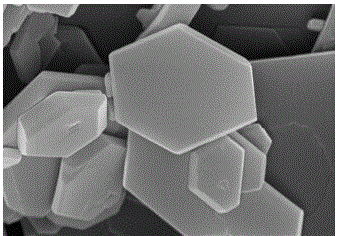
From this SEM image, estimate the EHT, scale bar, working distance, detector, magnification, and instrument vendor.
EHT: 15 kV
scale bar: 2000 nm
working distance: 8 mm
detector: SE(U)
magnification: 20 K X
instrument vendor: Hitachi